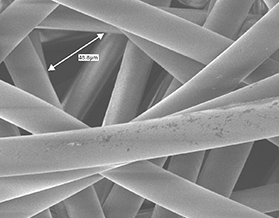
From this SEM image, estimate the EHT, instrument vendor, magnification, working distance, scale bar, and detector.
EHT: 2 kV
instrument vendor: JEOL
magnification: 0.55 K X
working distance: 10 mm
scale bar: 10000 nm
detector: SEI